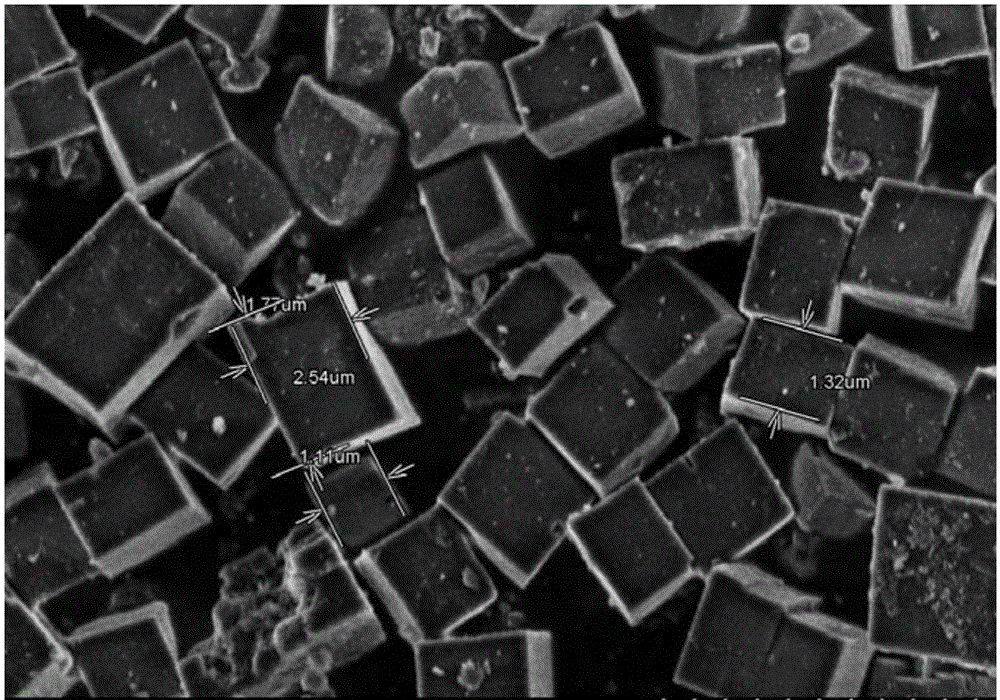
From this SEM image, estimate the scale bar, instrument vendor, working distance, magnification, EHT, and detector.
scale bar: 5000 nm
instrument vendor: Hitachi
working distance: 7.9 mm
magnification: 8 K X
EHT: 3 kV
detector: SE(M)